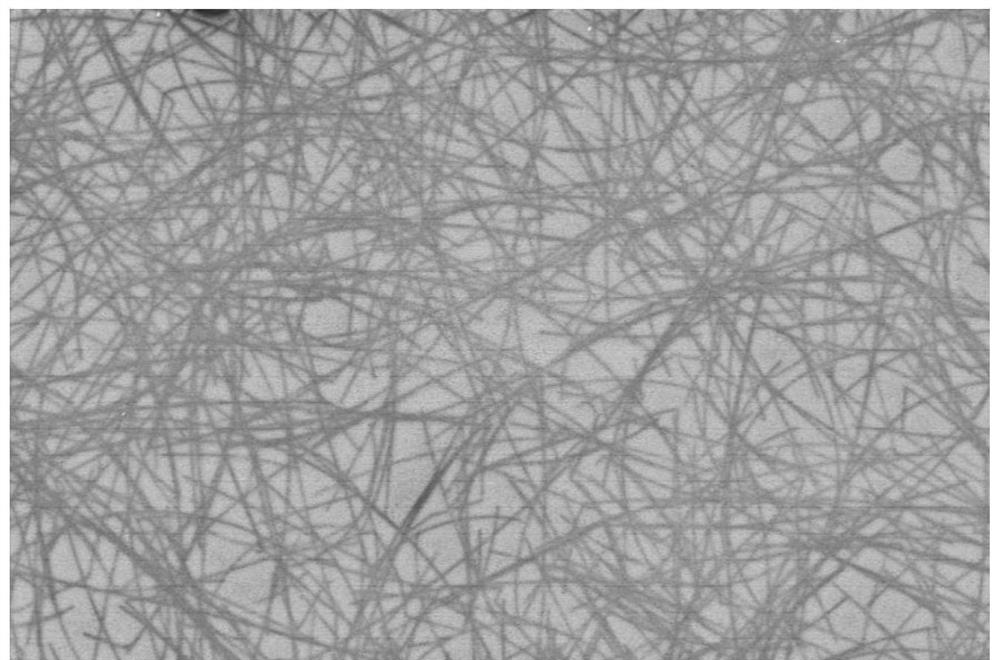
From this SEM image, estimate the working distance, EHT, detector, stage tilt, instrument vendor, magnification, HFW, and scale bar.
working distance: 4.2 mm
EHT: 2 kV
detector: TLD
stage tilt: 0°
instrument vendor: FEI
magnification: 8 K X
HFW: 51.8 µm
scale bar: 10000 nm